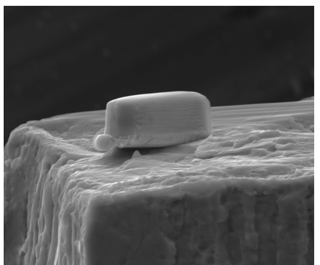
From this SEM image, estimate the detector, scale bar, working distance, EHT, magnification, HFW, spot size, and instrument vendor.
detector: ETD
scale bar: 5000 nm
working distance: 11.5 mm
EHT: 15 kV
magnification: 16.365 K X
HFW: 15.6 µm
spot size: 5.5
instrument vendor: FEI